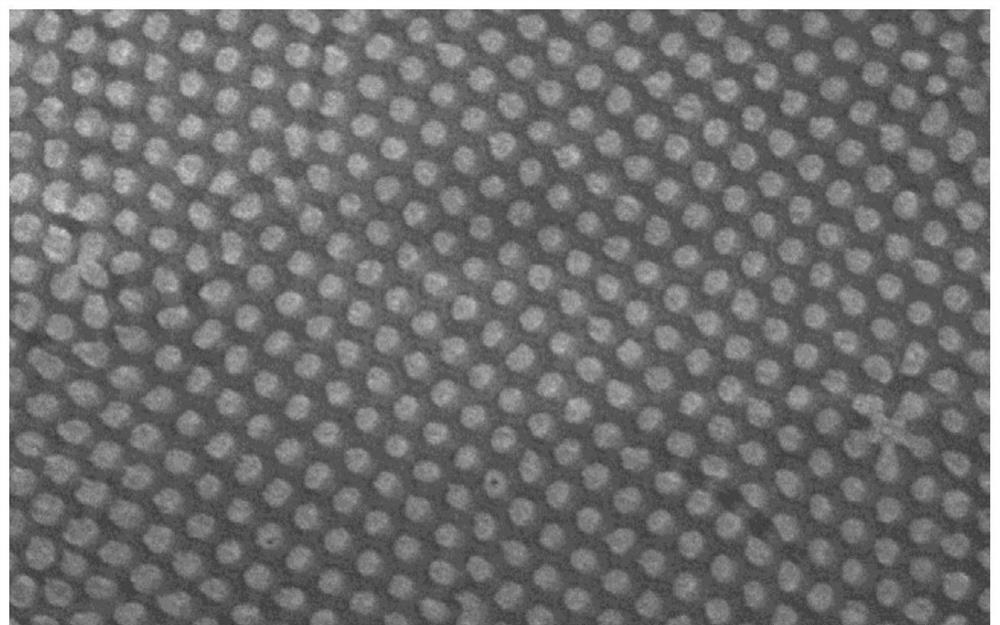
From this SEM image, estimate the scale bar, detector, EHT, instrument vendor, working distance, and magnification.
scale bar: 1000 nm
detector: SE(UL)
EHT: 3 kV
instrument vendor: Hitachi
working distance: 8.8 mm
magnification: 50 K X